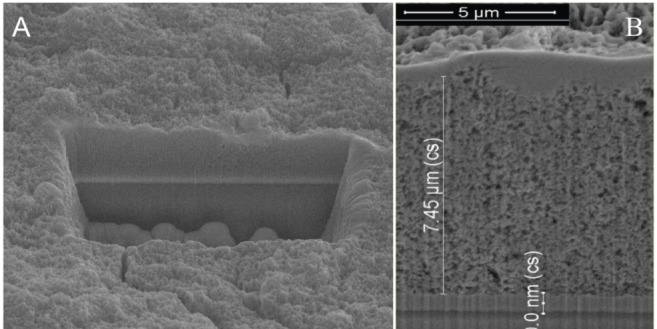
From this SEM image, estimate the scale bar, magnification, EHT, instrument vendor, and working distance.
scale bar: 10000 nm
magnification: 3.5 K X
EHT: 20 kV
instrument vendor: FEI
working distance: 10 mm